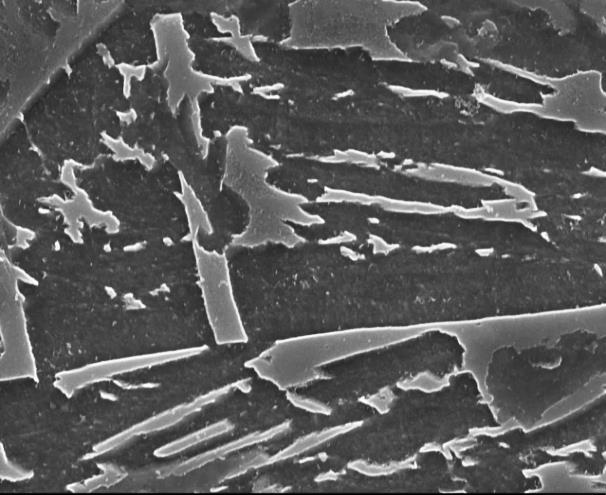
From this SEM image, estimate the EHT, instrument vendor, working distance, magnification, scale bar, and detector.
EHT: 20 kV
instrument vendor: JEOL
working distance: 24.3 mm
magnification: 10 K X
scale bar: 1000 nm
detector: SEI